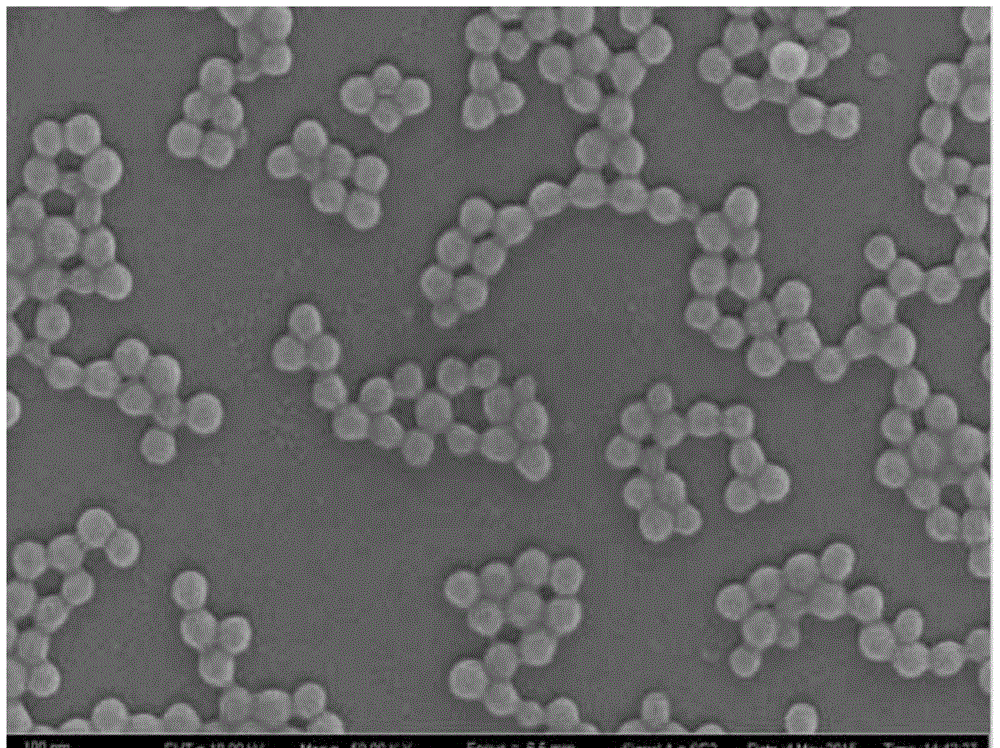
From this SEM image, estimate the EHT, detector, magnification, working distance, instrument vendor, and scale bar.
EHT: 10 kV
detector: SE2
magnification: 50 K X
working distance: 6.5 mm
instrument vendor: Zeiss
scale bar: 100 nm